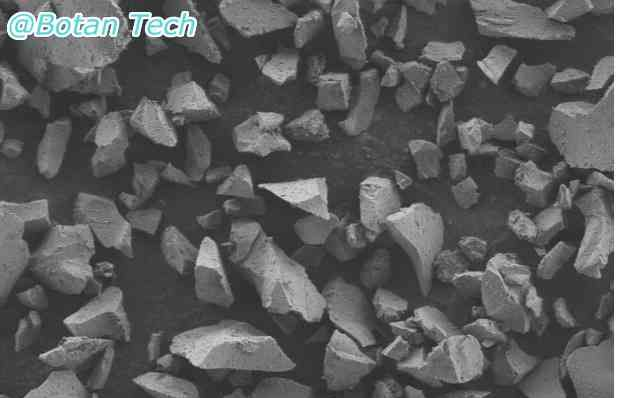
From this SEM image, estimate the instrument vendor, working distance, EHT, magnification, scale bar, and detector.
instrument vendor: Zeiss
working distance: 5 mm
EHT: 2 kV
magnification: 2 K X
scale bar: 20000 nm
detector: MPSE